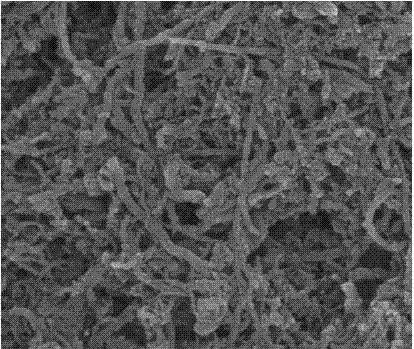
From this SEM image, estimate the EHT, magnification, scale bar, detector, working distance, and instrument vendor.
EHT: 15 kV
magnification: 100 K X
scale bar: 500 nm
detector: TLD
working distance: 5.7 mm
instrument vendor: FEI